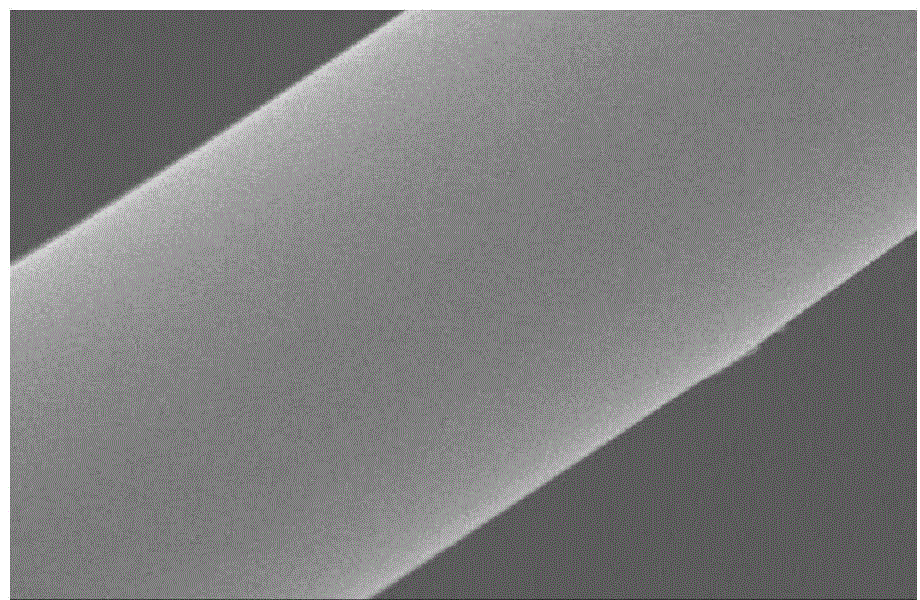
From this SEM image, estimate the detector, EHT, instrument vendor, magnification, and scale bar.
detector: SEI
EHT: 30 kV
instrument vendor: JEOL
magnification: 5 K X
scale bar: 5000 nm